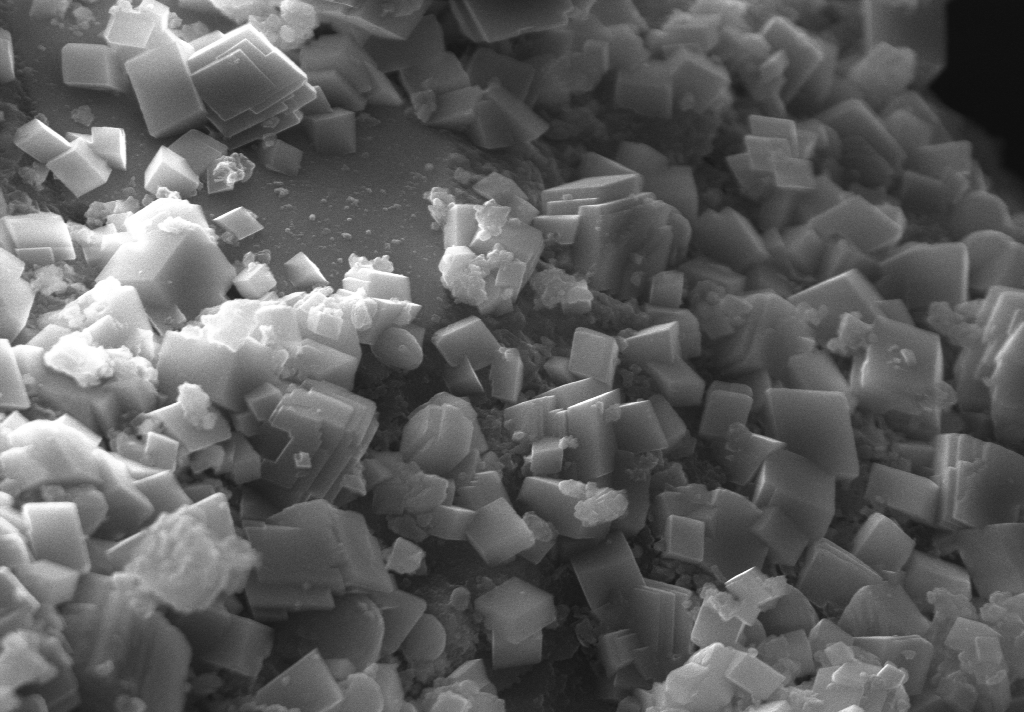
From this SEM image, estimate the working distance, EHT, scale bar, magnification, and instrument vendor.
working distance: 9.9 mm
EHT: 15 kV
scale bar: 5000 nm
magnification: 10 K X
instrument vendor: other